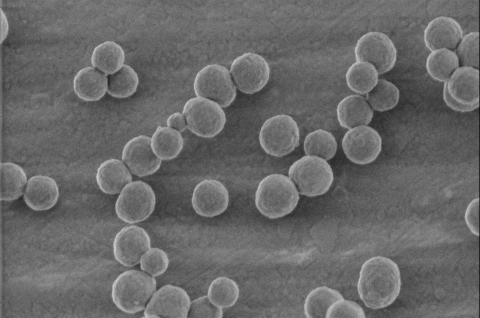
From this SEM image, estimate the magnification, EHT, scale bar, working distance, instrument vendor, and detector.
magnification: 50 K X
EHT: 0.5 kV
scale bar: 500 nm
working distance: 2.9 mm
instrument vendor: FEI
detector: TLD